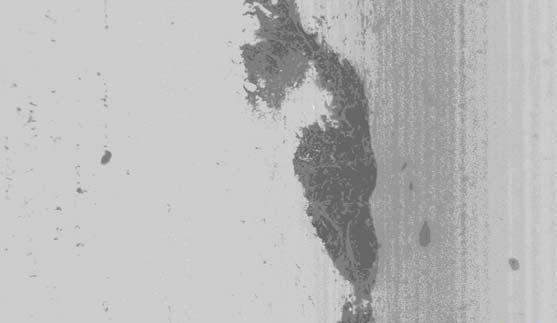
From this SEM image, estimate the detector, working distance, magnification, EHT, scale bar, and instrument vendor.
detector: CZ BSD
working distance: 16 mm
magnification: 0.075 K X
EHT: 25 kV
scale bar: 200000 nm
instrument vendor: Zeiss